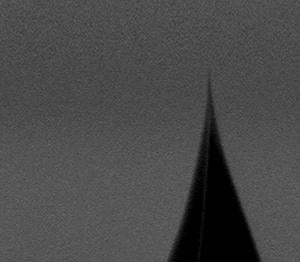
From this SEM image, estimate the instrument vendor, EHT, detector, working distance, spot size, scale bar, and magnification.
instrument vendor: FEI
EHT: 4 kV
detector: TLD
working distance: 4.7 mm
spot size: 3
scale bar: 200 nm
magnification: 80 K X